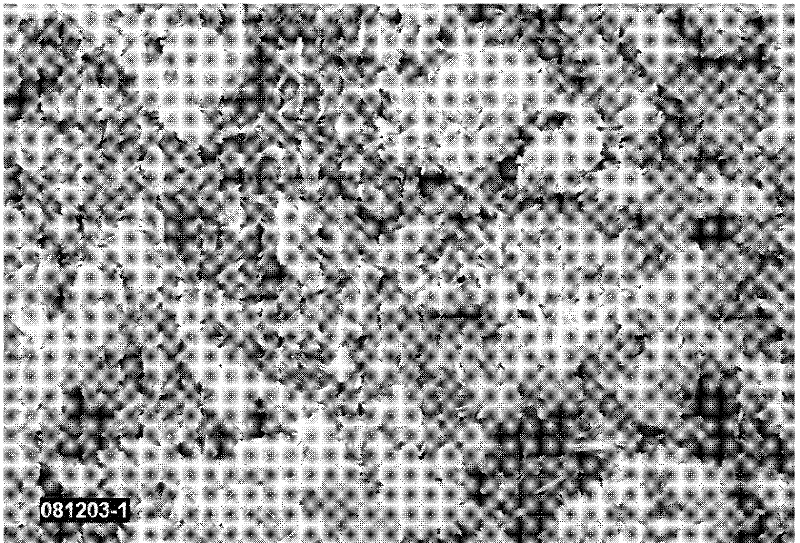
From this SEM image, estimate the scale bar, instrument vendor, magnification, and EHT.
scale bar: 10000 nm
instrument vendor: other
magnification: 1 K X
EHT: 20 kV